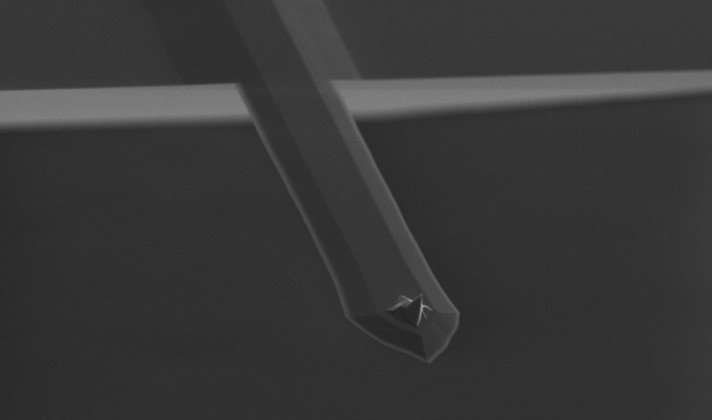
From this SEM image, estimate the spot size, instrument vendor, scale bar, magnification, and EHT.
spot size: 6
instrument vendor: FEI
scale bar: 50000 nm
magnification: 0.477 K X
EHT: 10 kV